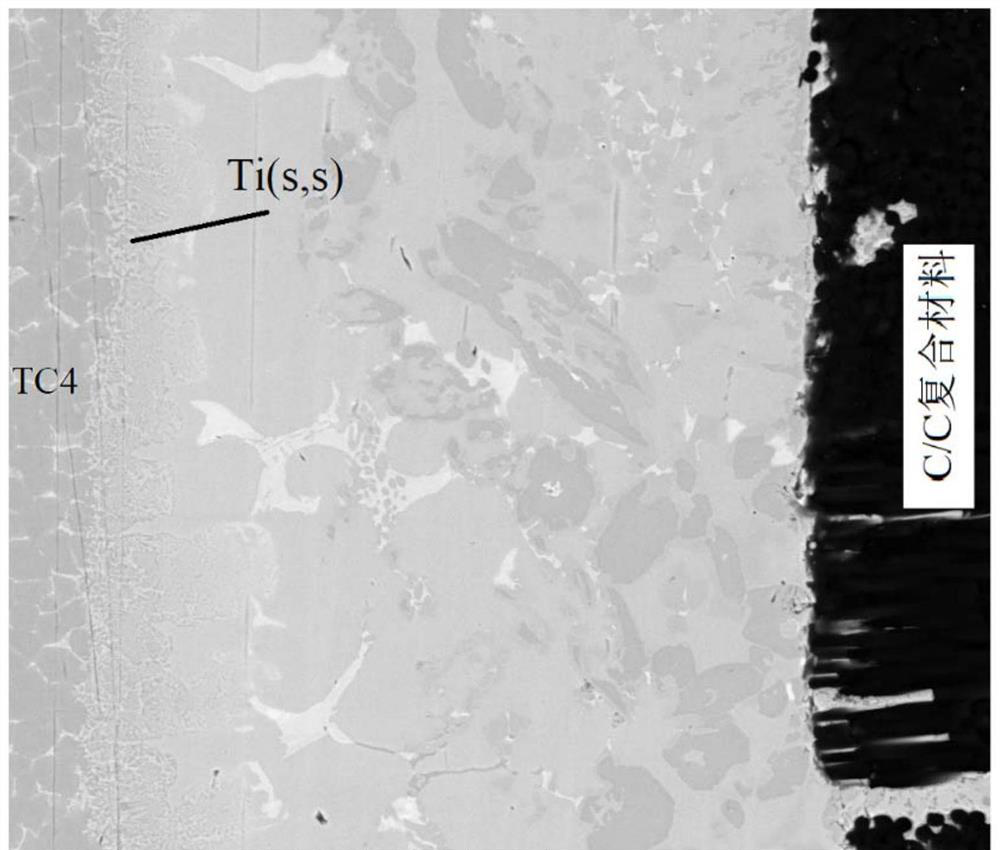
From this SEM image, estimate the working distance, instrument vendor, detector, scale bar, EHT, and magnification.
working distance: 9.4 mm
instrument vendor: FEI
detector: Z Cont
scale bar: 100000 nm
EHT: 30 kV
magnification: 0.8 K X